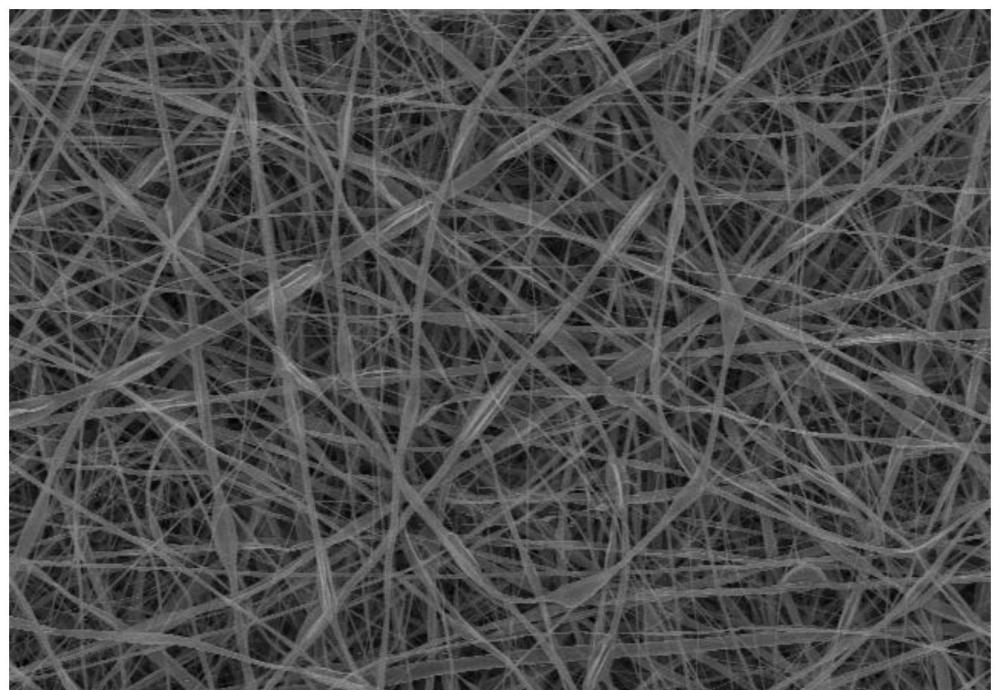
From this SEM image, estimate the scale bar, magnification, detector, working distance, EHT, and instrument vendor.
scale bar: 50000 nm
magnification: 1 K X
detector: SE(U)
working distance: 19.6 mm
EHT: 10 kV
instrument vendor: Hitachi